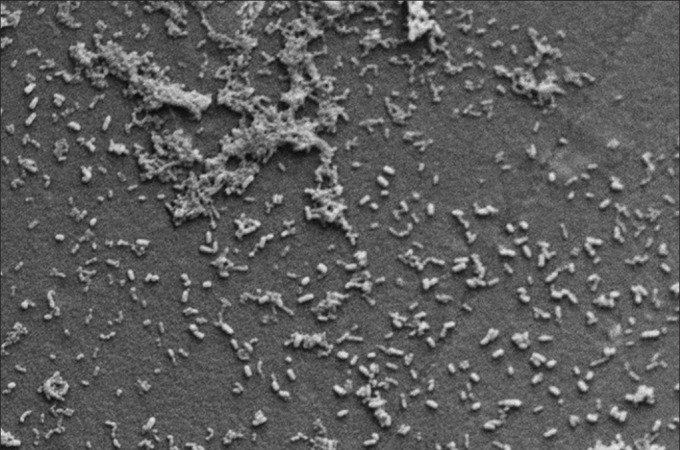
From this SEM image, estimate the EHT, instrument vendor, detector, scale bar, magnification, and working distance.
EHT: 15 kV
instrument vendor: Zeiss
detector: SE1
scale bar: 3000 nm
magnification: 1.29 K X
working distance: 18 mm